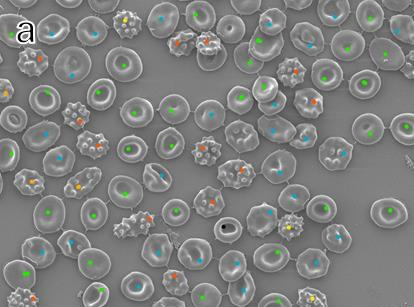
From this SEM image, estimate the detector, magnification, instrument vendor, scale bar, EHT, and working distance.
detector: LED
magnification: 1.5 K X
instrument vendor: JEOL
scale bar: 10000 nm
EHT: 2 kV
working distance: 10 mm